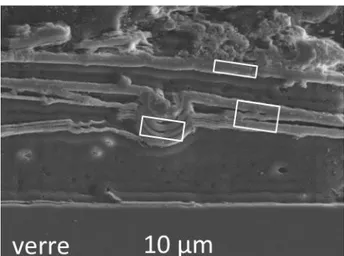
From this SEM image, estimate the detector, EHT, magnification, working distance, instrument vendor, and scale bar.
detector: SEI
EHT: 15 kV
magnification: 0.9 K X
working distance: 10 mm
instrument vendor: JEOL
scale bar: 10000 nm